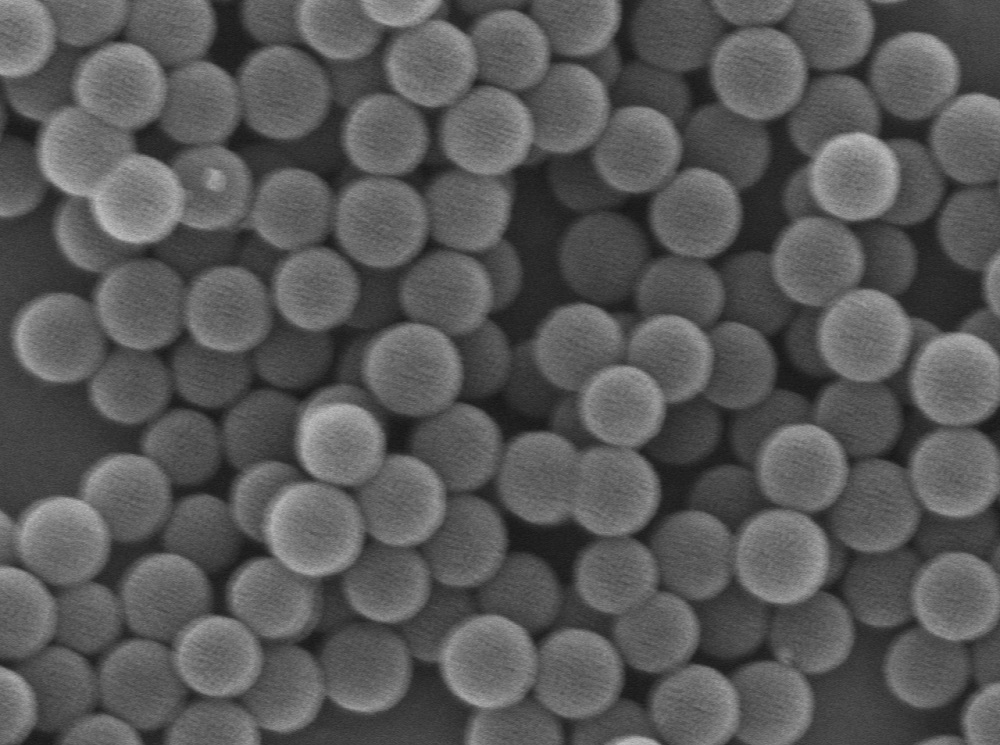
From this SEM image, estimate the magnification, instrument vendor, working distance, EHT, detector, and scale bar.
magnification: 55 K X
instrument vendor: JEOL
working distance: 6 mm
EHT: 5 kV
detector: SEI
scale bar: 100 nm